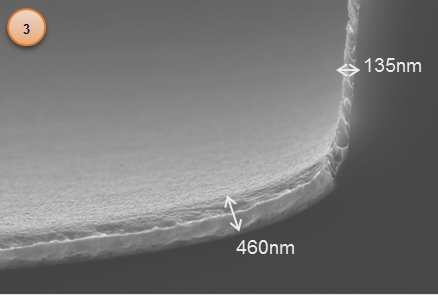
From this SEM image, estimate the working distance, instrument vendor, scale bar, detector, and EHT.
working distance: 3.9 mm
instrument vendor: Zeiss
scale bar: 200 nm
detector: InLens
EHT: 10 kV